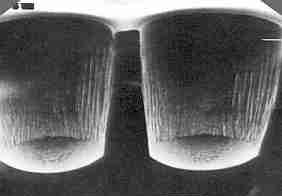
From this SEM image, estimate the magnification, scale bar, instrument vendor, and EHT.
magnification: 0.35 K X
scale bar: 50000 nm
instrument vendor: JEOL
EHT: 10 kV